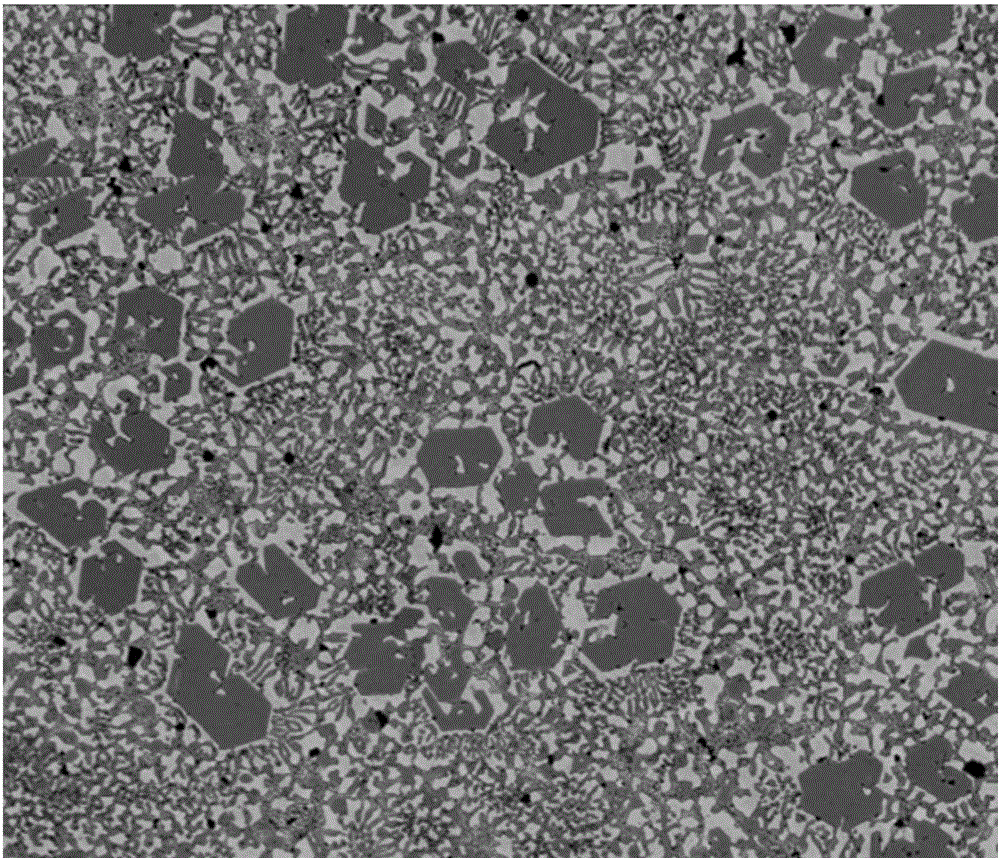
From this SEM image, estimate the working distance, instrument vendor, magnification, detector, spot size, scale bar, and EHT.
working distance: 9.6 mm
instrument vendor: FEI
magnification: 0.5 K X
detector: SSD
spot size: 7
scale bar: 50000 nm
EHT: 15 kV